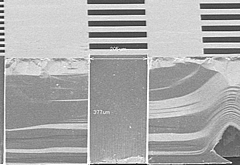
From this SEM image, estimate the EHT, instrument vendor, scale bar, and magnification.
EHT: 20 kV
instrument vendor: Hitachi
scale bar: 300000 nm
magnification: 0.15 K X